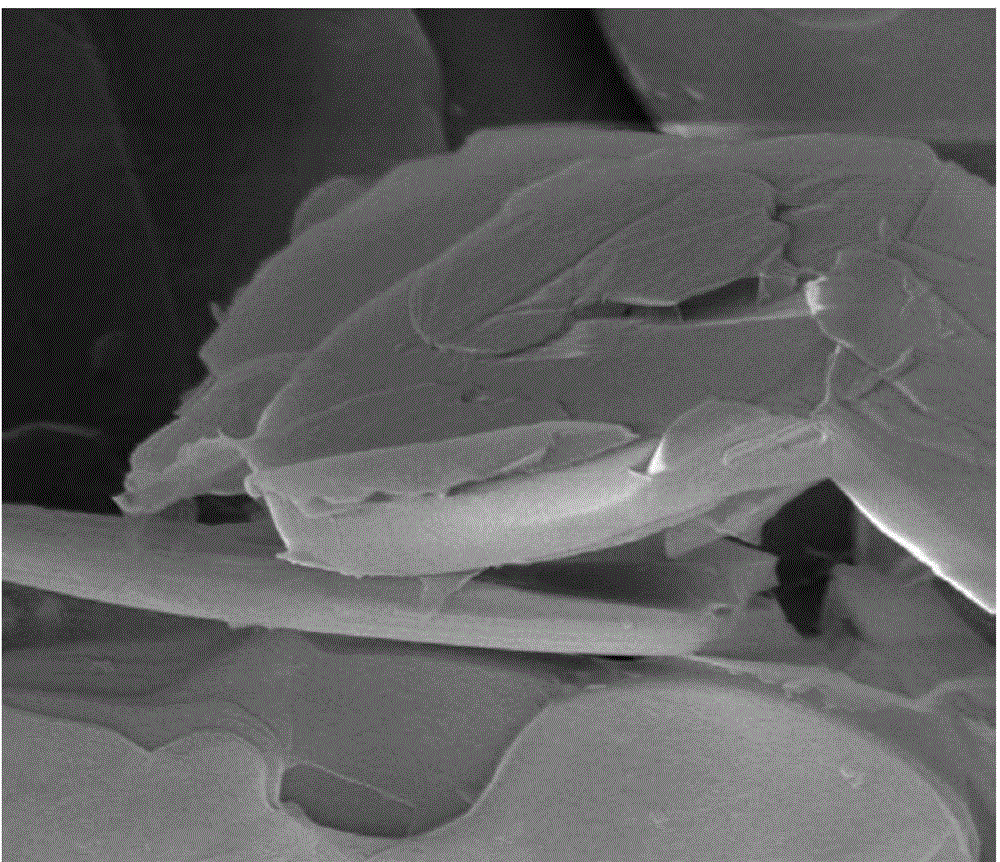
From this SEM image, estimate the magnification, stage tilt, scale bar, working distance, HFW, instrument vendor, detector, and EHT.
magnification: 30 K X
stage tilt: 0°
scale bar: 4000 nm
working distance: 12.1 mm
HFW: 9.95 µm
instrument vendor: FEI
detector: ETD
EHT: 10 kV